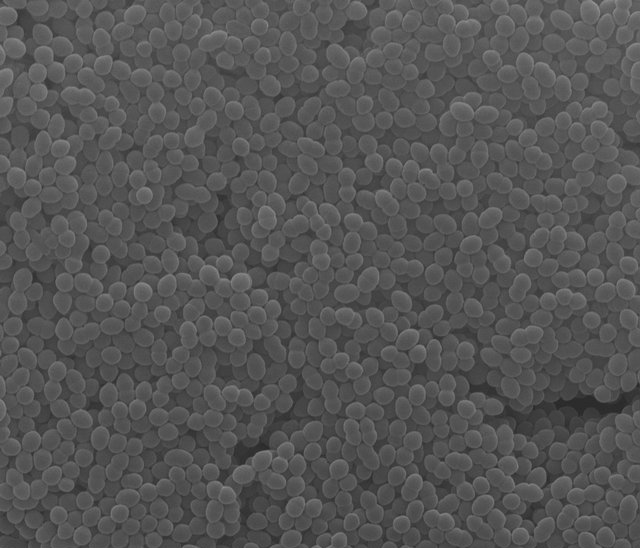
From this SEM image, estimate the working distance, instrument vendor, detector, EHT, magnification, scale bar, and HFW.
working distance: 11.4 mm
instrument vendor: FEI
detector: ETD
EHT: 20 kV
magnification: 5 K X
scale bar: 5000 nm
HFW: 27.04 µm